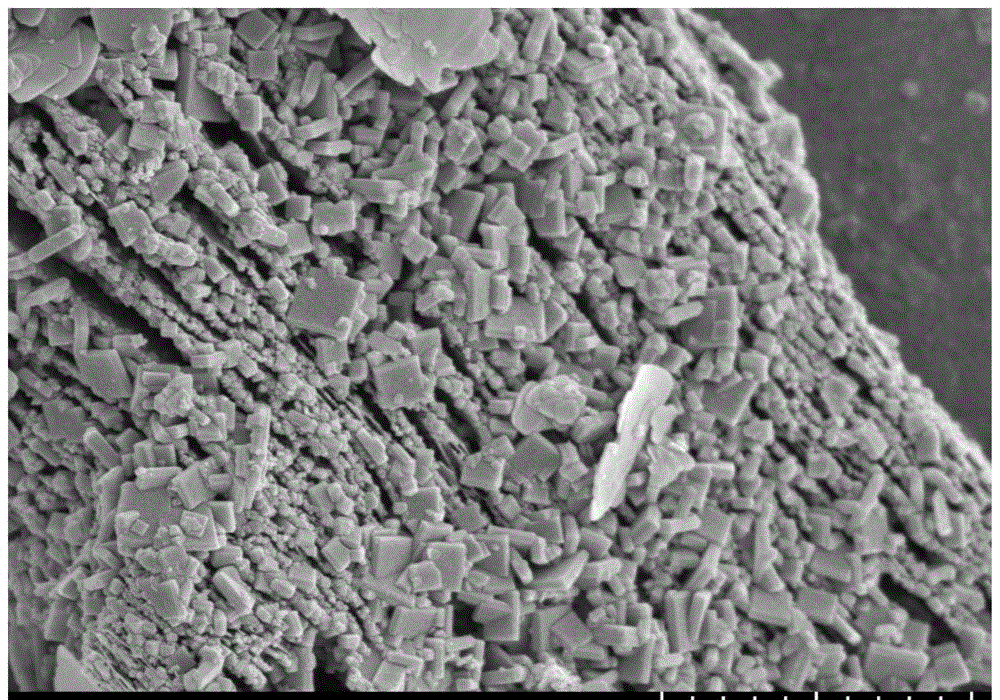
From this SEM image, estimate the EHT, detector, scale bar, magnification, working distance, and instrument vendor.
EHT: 3 kV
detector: SE(UL)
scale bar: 2000 nm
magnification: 20 K X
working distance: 8.9 mm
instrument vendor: Hitachi